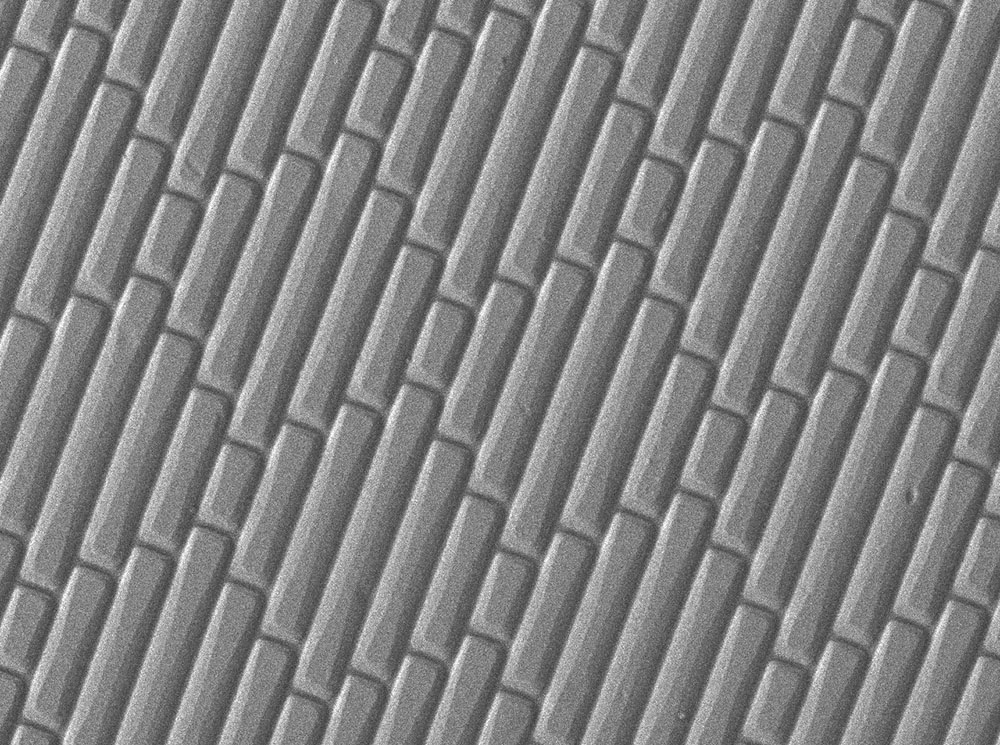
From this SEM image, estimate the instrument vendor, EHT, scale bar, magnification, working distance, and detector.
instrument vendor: JEOL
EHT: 2 kV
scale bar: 10000 nm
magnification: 0.5 K X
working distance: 10.7 mm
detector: LEI